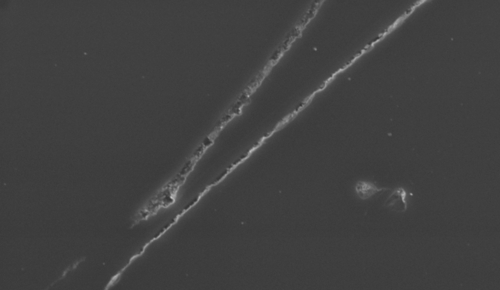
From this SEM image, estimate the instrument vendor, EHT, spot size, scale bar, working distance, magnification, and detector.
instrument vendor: FEI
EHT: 15 kV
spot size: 6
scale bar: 20000 nm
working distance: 20.2 mm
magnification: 2 K X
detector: SE